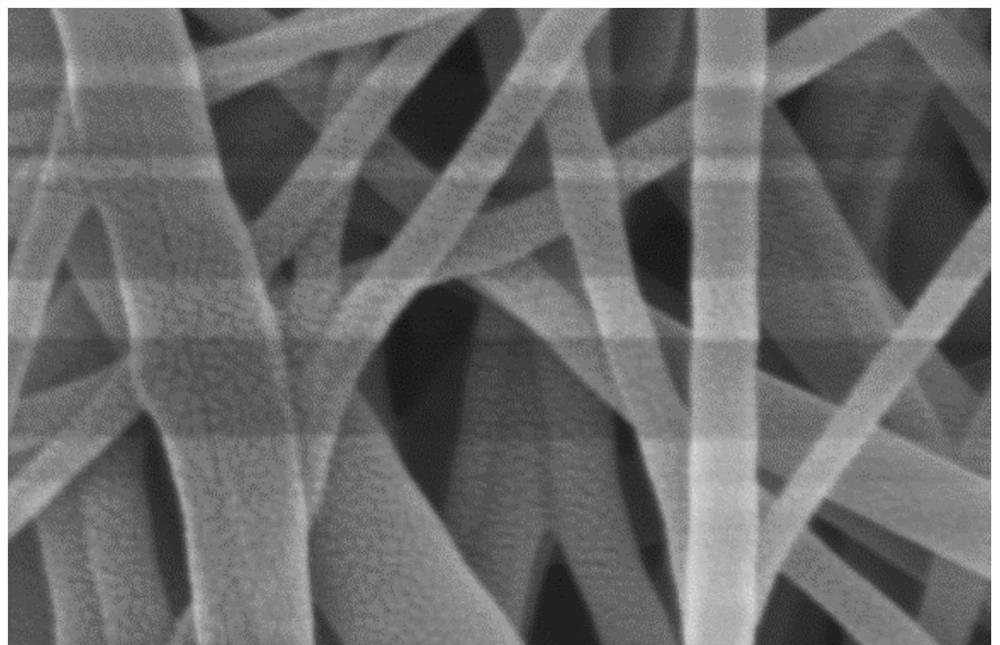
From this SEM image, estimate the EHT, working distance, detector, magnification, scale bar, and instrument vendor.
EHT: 5 kV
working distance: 8.2 mm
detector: SE(M)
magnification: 60 K X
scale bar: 500 nm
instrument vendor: Hitachi